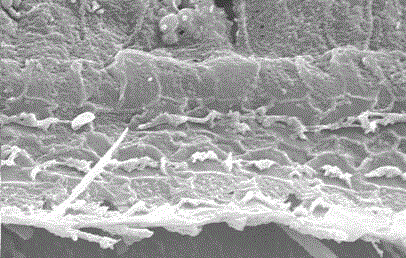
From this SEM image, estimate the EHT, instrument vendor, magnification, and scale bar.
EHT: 20 kV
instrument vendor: JEOL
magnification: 1.5 K X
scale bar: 5500 nm